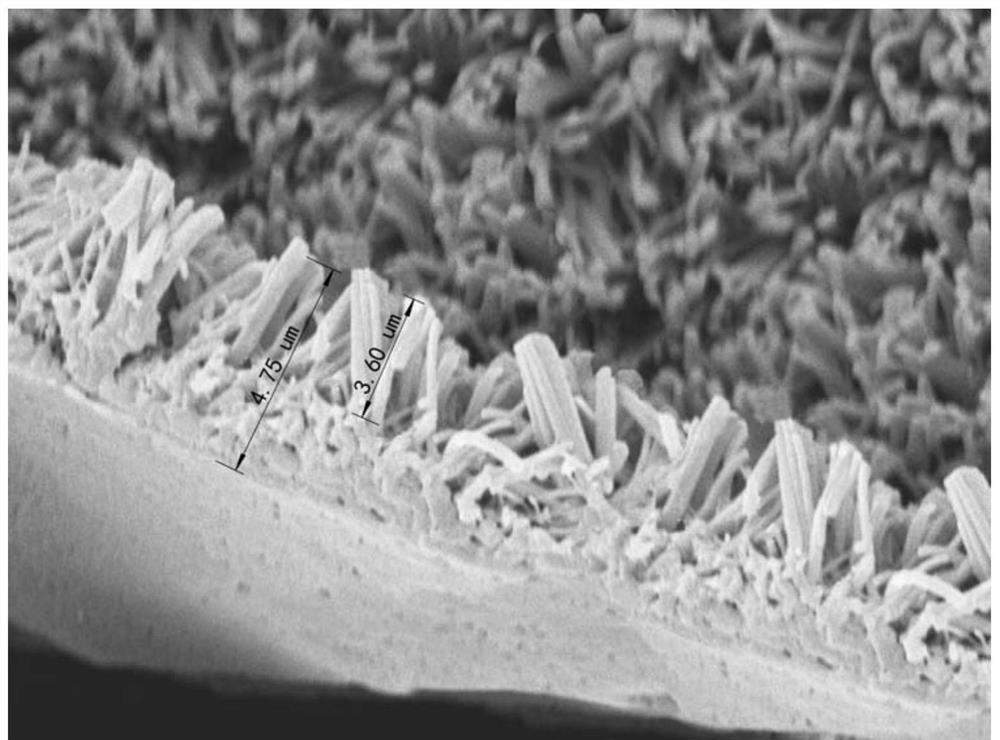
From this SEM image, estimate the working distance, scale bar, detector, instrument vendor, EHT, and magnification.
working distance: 12.8 mm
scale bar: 5000 nm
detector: SE(M,LA0)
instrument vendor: Hitachi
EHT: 5 kV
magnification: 6 K X